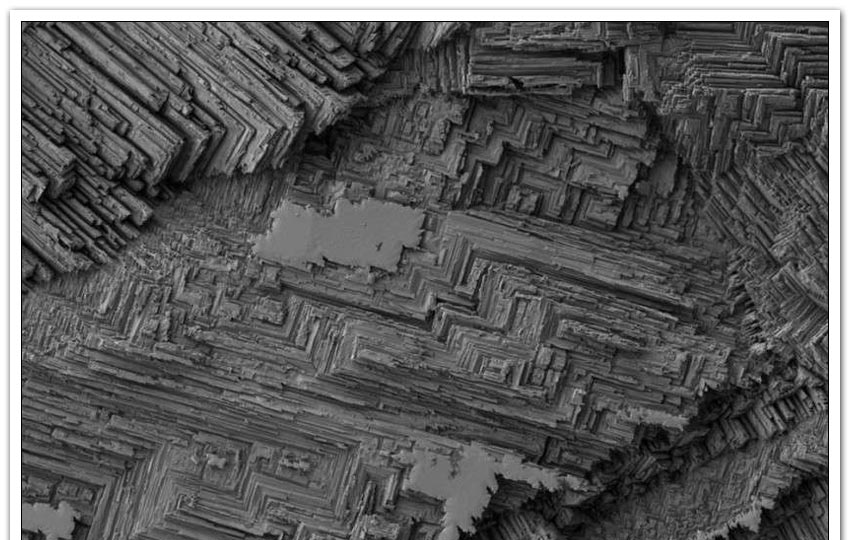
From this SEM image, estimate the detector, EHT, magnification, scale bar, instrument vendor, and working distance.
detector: SE2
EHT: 1 kV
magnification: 5 K X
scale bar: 1000 nm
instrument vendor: Zeiss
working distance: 4.8 mm